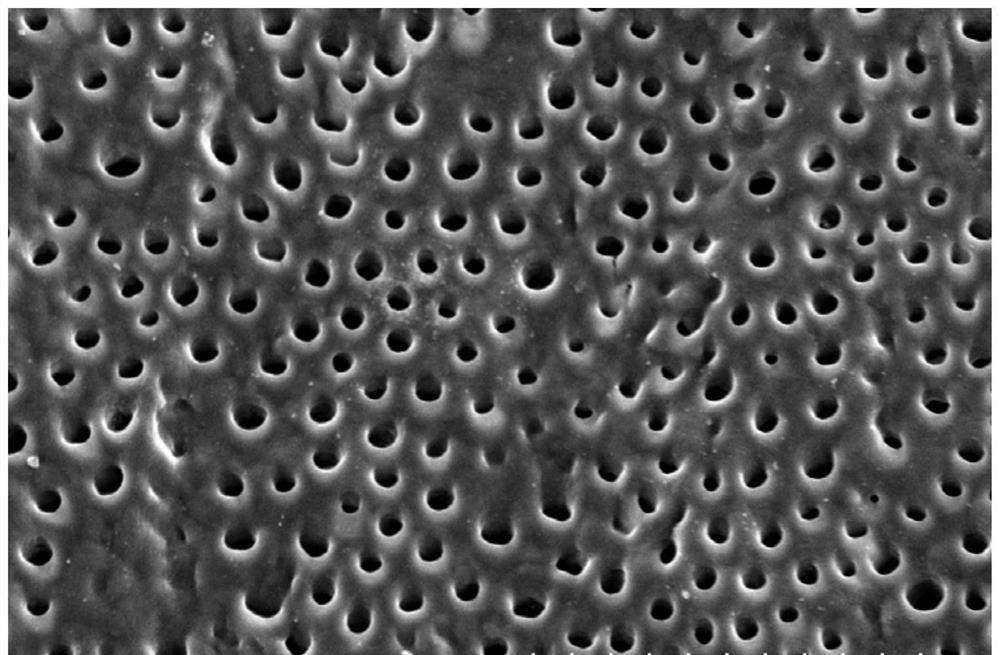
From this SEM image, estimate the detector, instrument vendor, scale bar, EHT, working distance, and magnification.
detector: SE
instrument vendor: Hitachi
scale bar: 50000 nm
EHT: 10 kV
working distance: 12.4 mm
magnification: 1 K X